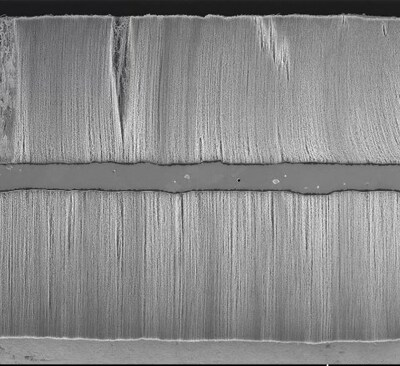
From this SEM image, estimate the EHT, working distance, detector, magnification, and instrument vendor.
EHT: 2 kV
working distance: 11.7 mm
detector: SE(U)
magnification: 0.45 K X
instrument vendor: Hitachi